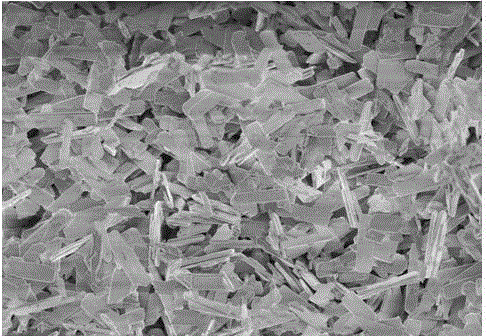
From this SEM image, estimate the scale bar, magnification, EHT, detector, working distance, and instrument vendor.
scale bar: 20000 nm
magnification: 2 K X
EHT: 1 kV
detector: SE(M,LA0)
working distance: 8.1 mm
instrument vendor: Hitachi